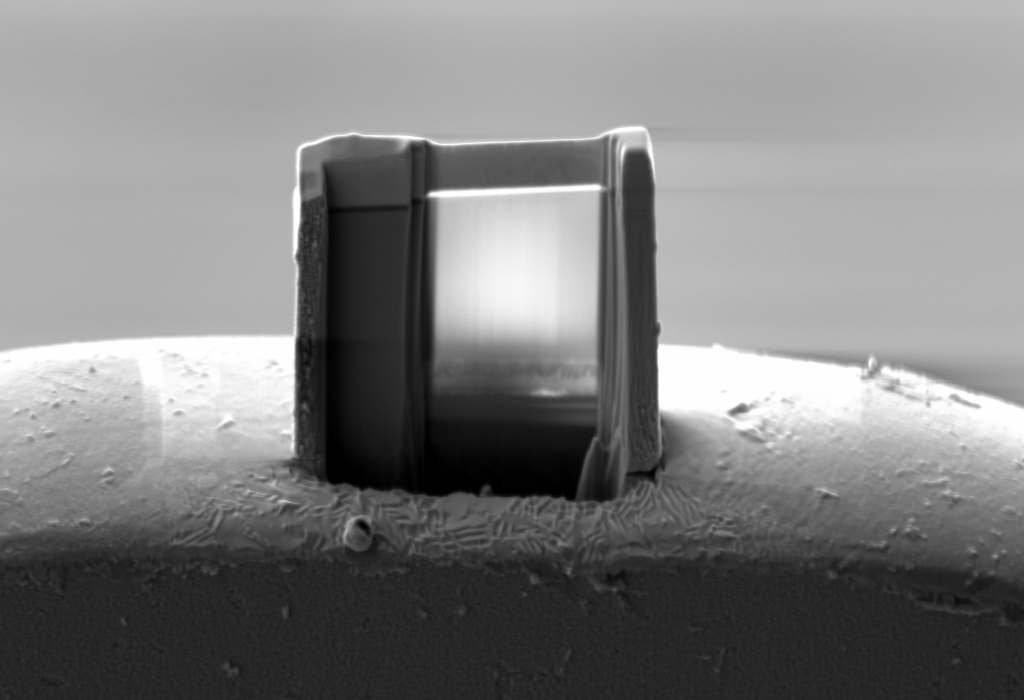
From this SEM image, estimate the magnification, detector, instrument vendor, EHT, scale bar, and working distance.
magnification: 3.92 K X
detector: SE2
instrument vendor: Zeiss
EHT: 5 kV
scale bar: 1000 nm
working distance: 5.2 mm